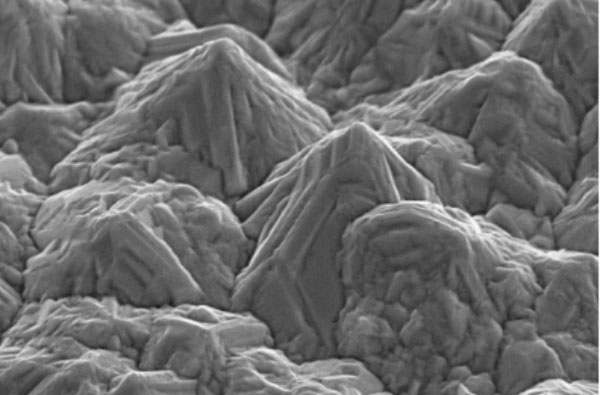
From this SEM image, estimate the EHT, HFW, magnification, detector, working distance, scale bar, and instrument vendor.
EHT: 10 kV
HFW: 12.81 µm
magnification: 10 K X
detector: ETD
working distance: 3.61 mm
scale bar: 1000 nm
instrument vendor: FEI